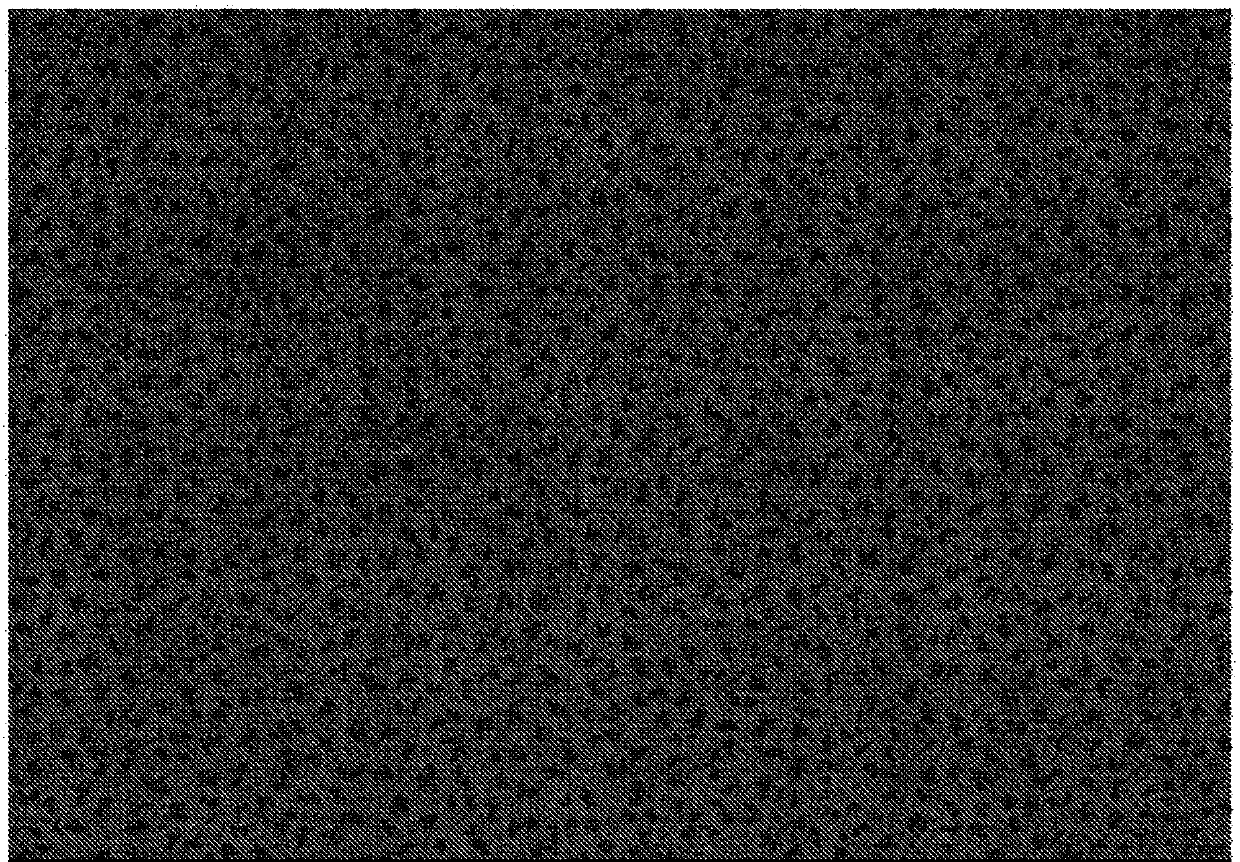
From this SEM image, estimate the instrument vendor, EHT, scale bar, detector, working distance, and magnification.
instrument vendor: Hitachi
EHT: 20 kV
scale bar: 50000 nm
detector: SE(M,LA3)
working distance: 9 mm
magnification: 1 K X